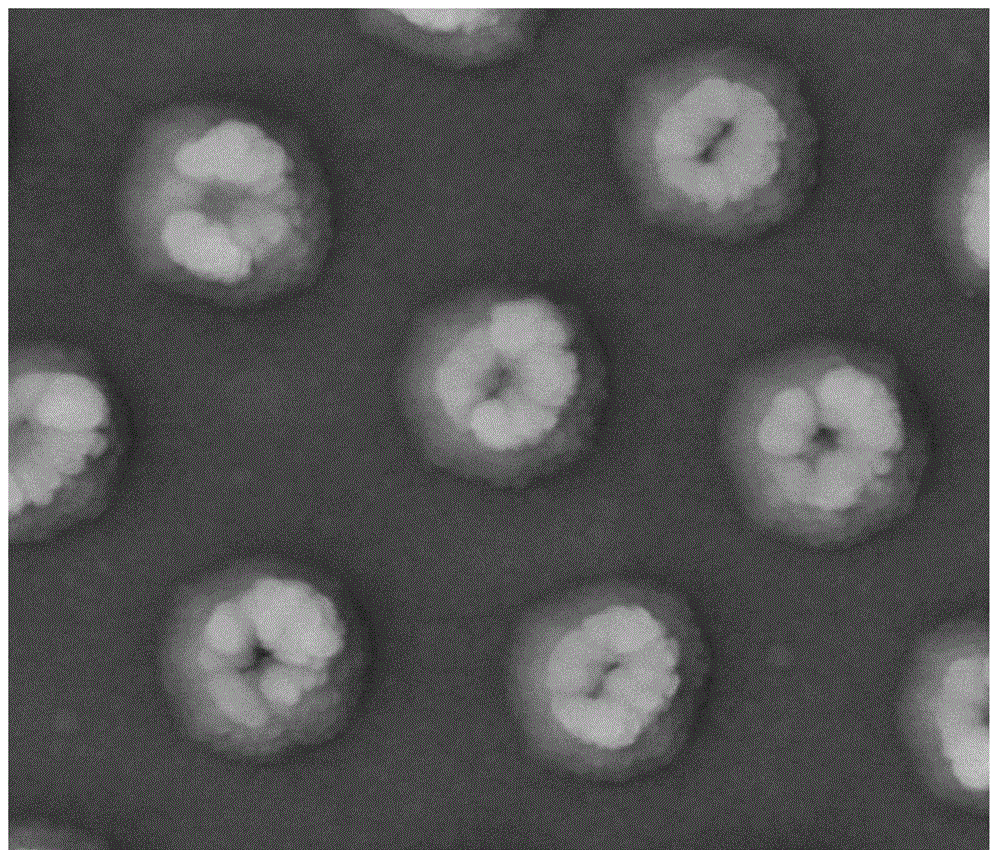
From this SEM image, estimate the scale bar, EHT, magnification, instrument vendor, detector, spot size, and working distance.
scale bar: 1000 nm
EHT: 20 kV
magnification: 100 K X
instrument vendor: FEI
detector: LFD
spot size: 3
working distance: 10.1 mm